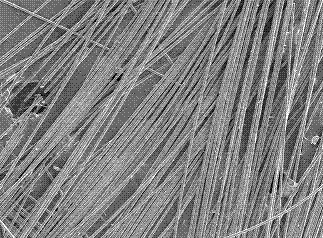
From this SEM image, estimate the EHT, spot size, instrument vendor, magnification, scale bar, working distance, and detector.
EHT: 10 kV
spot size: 3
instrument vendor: FEI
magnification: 0.1 K X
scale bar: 200000 nm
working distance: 11.6 mm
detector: SE